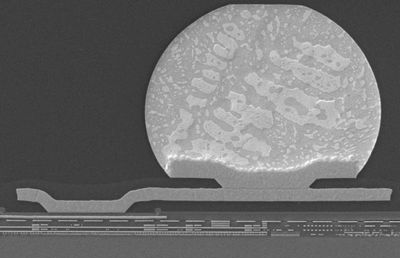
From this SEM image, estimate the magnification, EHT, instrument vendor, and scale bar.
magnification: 0.8 K X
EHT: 15 kV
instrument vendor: JEOL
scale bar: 20000 nm